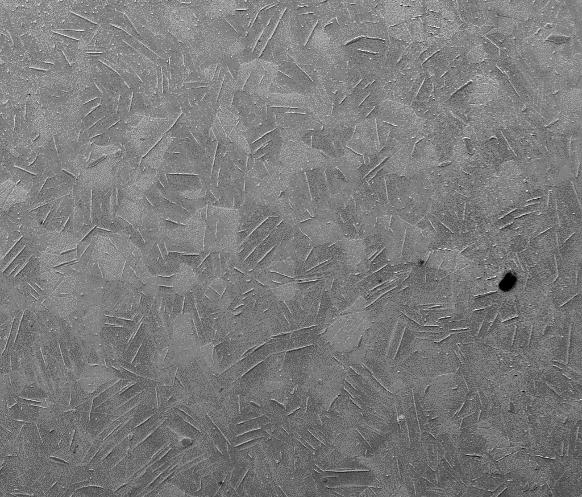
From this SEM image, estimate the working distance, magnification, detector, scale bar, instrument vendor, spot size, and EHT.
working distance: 10.7 mm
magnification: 0.1 K X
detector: Mix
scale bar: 500000 nm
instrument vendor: FEI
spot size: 4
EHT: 15 kV